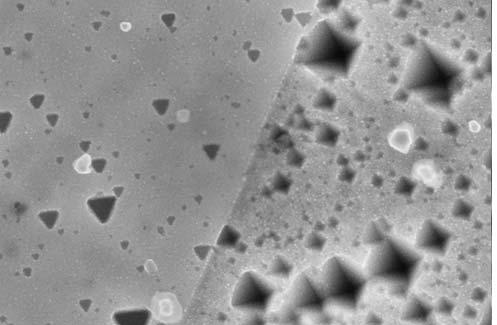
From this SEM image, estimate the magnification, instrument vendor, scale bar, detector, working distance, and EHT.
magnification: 25 K X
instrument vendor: Zeiss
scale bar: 200 nm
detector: InLens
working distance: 3.5 mm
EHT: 15 kV